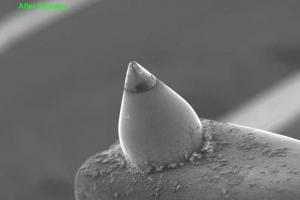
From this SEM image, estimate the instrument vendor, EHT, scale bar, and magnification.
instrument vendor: JEOL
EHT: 15 kV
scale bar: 200000 nm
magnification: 0.09 K X